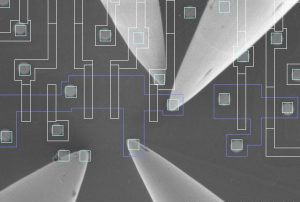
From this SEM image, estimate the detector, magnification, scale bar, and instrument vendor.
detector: SE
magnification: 15 K X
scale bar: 3000 nm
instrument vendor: Hitachi